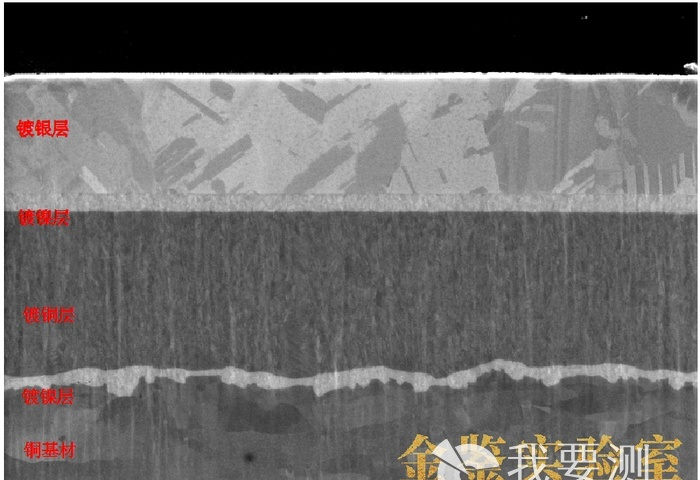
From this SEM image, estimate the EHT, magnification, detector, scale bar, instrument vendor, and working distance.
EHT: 5 kV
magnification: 12 K X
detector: InLens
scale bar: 1000 nm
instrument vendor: Zeiss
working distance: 4.6 mm